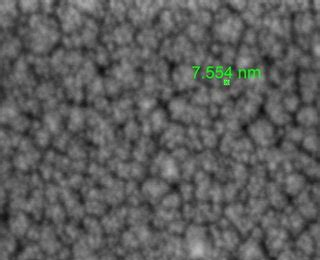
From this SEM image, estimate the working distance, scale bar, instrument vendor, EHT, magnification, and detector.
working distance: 3.2 mm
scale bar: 300 nm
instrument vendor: FEI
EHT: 2 kV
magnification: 500 K X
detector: TLD